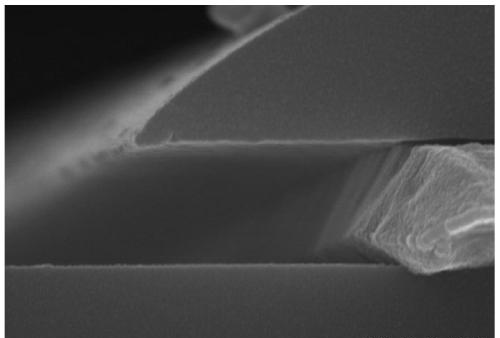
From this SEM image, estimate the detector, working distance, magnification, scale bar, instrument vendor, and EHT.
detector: SE(M)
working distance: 9.1 mm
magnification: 60 K X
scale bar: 500 nm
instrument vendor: Hitachi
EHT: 15 kV